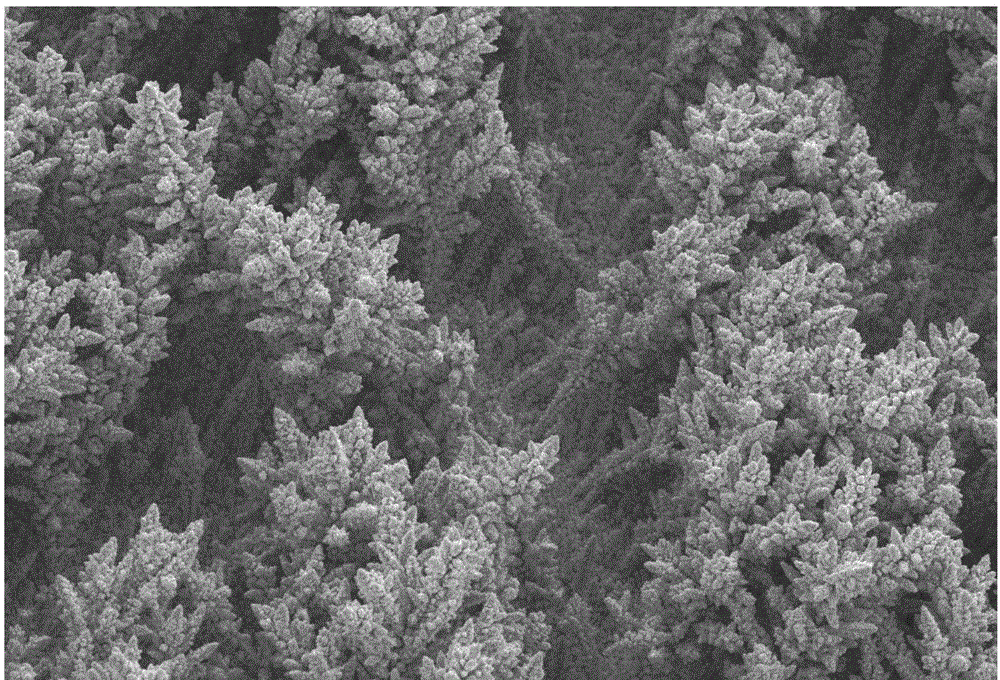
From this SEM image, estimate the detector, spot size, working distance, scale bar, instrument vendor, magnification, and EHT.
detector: SEI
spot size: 45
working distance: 28 mm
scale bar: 100000 nm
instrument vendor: JEOL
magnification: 0.25 K X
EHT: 30 kV